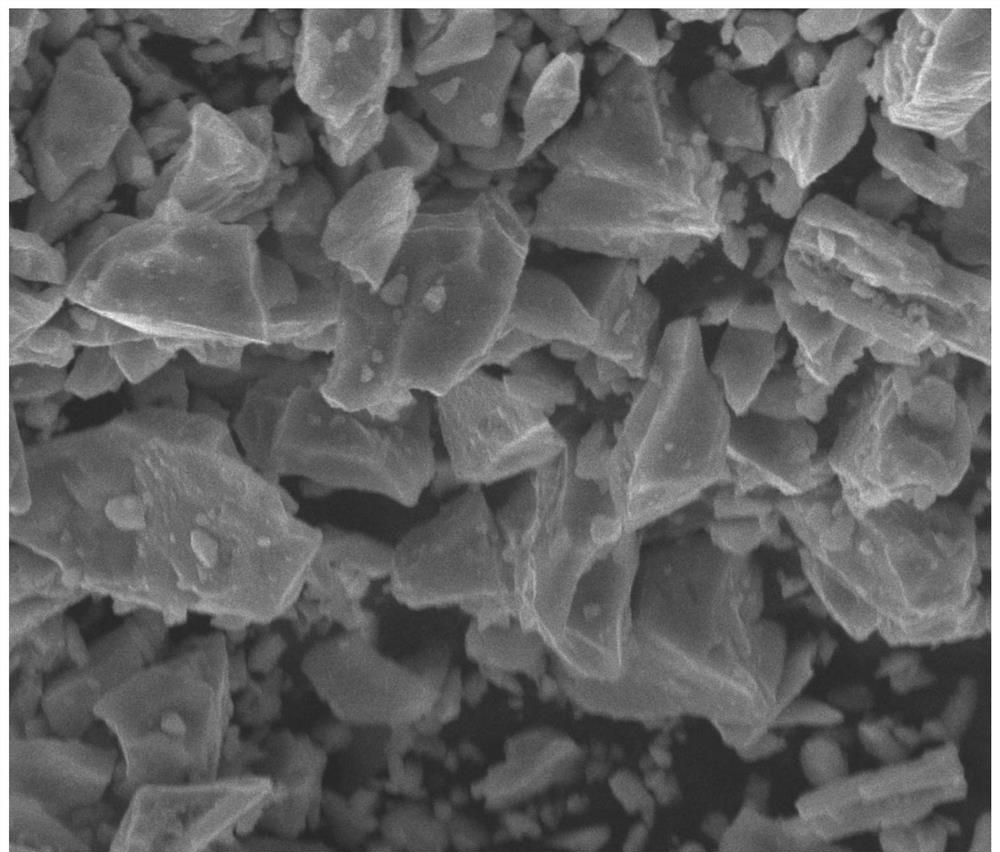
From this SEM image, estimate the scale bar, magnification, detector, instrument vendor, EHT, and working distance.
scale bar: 10000 nm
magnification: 4 K X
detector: ETD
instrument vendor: FEI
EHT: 25 kV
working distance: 24.1 mm